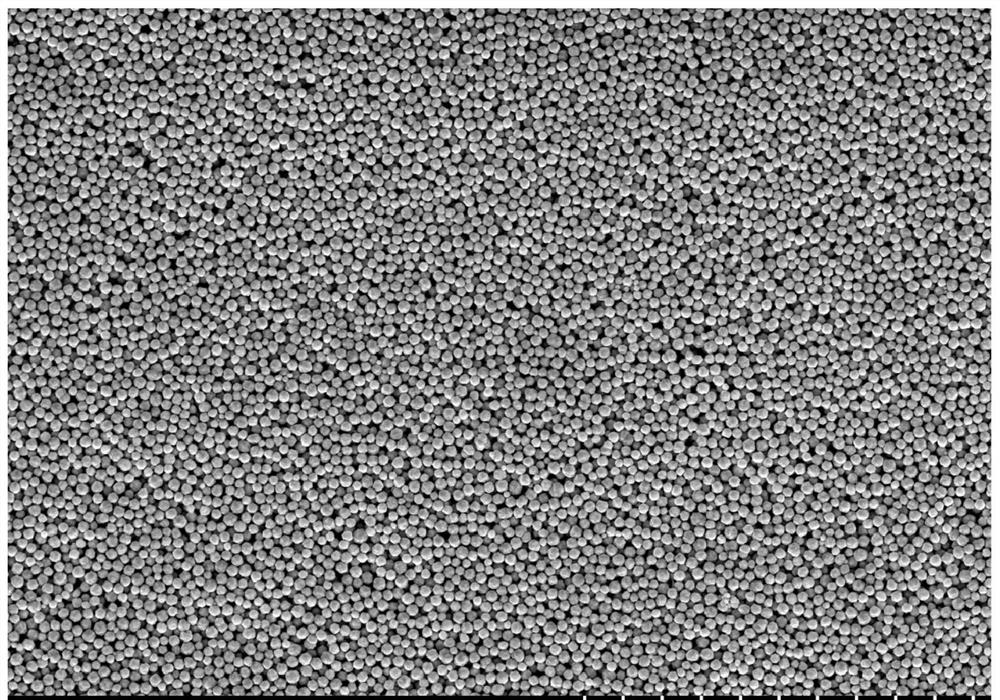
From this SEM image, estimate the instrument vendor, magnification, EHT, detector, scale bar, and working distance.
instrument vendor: Hitachi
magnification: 50 K X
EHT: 3 kV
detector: SE(M)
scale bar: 1000 nm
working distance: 7.5 mm